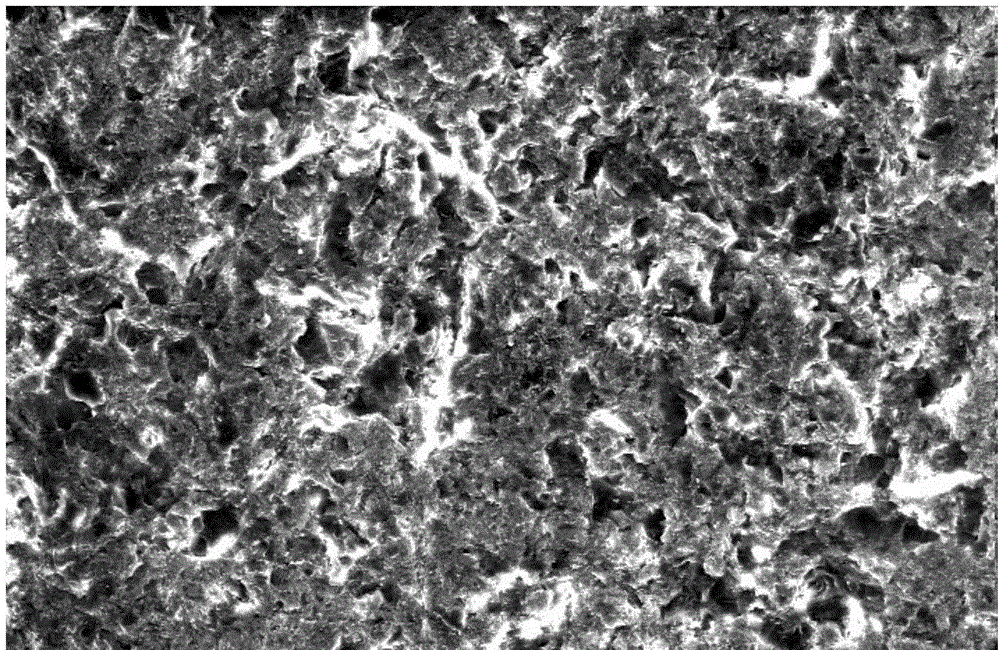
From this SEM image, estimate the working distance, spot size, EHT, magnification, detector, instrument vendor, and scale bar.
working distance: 12.2 mm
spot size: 4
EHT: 20 kV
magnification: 0.5 K X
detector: GSE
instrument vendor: FEI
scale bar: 100000 nm